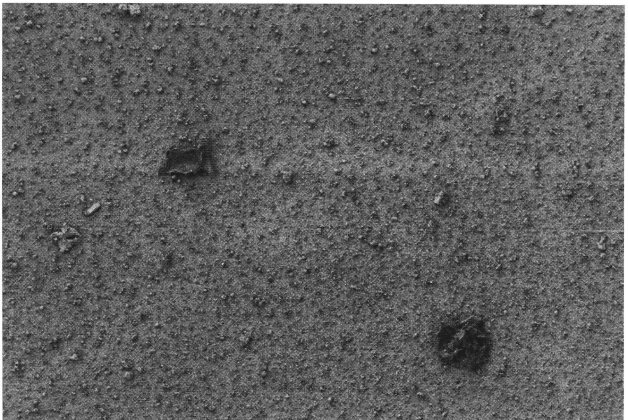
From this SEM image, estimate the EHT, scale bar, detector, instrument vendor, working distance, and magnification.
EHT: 1 kV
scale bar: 20000 nm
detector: SE2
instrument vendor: Zeiss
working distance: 5 mm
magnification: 1 K X